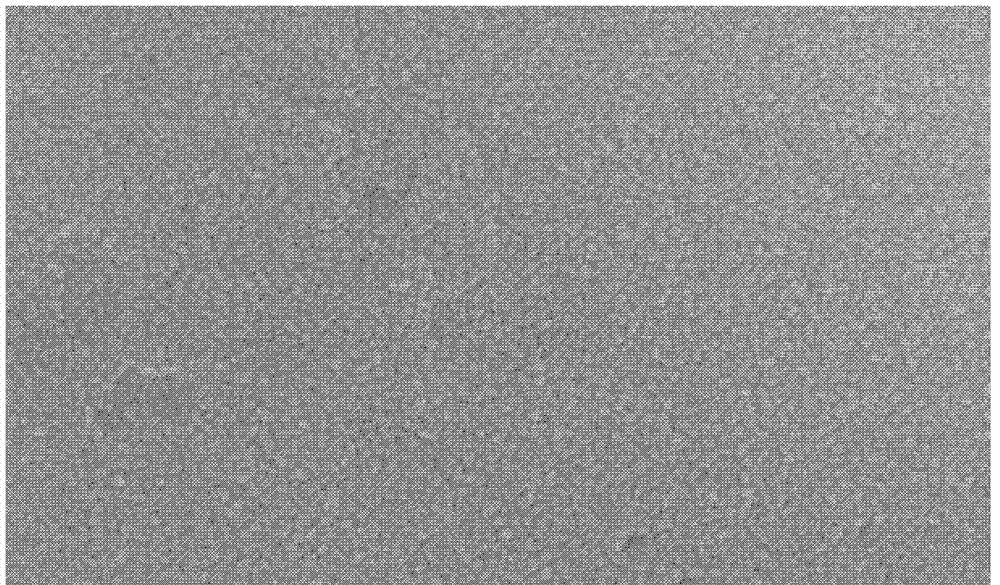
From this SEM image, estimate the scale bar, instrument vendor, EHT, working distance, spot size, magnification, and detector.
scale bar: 500 nm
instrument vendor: FEI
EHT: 15 kV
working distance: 8.1 mm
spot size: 3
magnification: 40 K X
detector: SE